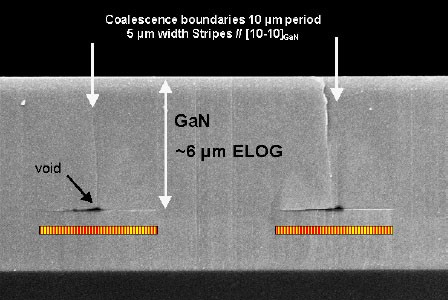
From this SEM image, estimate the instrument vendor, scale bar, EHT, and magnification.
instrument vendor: JEOL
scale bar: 1000 nm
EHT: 15 kV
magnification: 6 K X